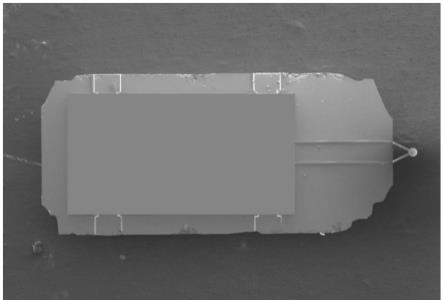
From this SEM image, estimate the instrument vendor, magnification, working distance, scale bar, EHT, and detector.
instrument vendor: Hitachi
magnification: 0.03 K X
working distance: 9 mm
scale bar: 1000000 nm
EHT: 3 kV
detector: LM(UL)